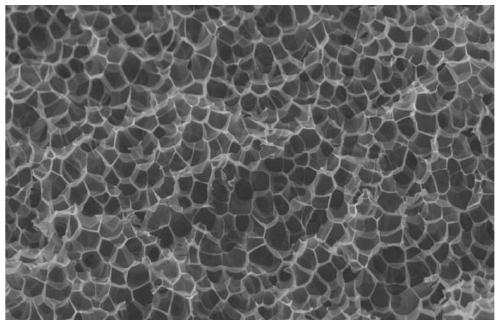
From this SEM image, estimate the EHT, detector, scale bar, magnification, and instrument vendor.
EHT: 12 kV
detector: SEI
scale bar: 100000 nm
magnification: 0.2 K X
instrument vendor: JEOL